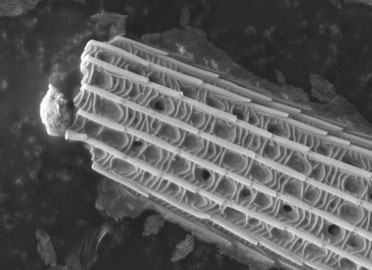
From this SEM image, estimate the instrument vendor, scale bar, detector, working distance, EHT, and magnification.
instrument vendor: JEOL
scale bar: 1000 nm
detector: LEI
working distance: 20.2 mm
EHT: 5 kV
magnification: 10 K X